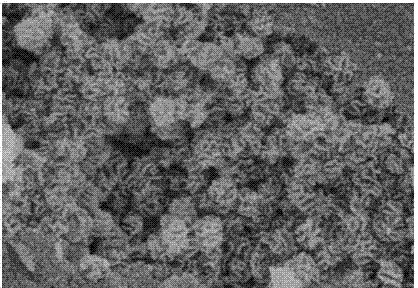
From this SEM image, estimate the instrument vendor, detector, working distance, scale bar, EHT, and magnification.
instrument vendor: Hitachi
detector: SE(UL)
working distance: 6 mm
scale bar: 500 nm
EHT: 3 kV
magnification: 100 K X